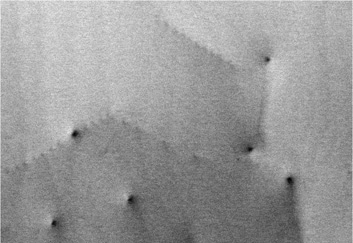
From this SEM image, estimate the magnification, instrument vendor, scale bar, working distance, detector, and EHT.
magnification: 100 K X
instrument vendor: Hitachi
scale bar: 500 nm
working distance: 4.8 mm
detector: BSE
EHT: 15 kV